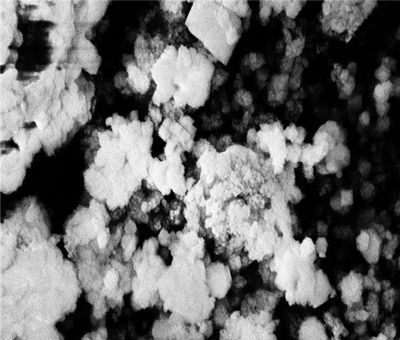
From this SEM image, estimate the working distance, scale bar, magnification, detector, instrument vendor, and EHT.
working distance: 6.3 mm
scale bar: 200 nm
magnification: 33.15 K X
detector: InLens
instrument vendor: Zeiss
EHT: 5 kV